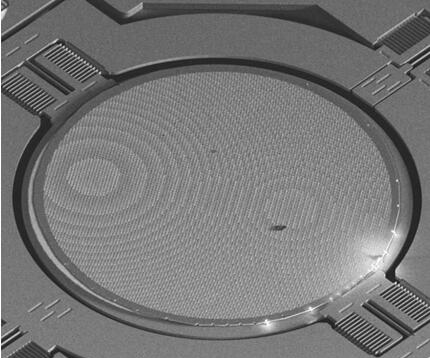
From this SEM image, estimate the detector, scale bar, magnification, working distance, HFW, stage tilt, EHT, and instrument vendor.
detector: ETD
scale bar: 300000 nm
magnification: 0.13 K X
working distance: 5.2 mm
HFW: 985 µm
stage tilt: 45°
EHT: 5 kV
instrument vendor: FEI